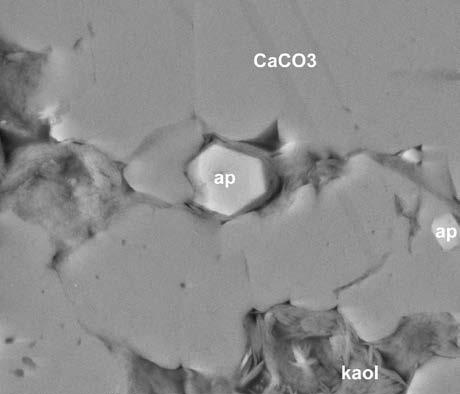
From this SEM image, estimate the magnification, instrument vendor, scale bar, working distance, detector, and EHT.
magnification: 16 K X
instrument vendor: FEI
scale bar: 10000 nm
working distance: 9.6 mm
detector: BSE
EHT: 15 kV